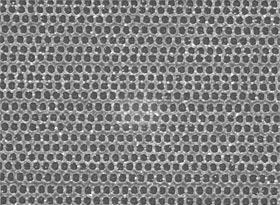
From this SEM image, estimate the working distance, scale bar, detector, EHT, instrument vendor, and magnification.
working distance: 7 mm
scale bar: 1000 nm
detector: SEI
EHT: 12 kV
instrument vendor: JEOL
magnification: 20 K X